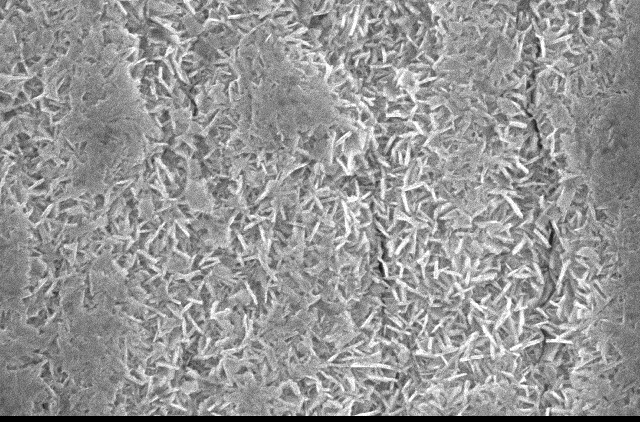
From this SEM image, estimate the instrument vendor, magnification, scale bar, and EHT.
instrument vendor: JEOL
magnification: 10 K X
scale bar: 1000 nm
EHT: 20 kV